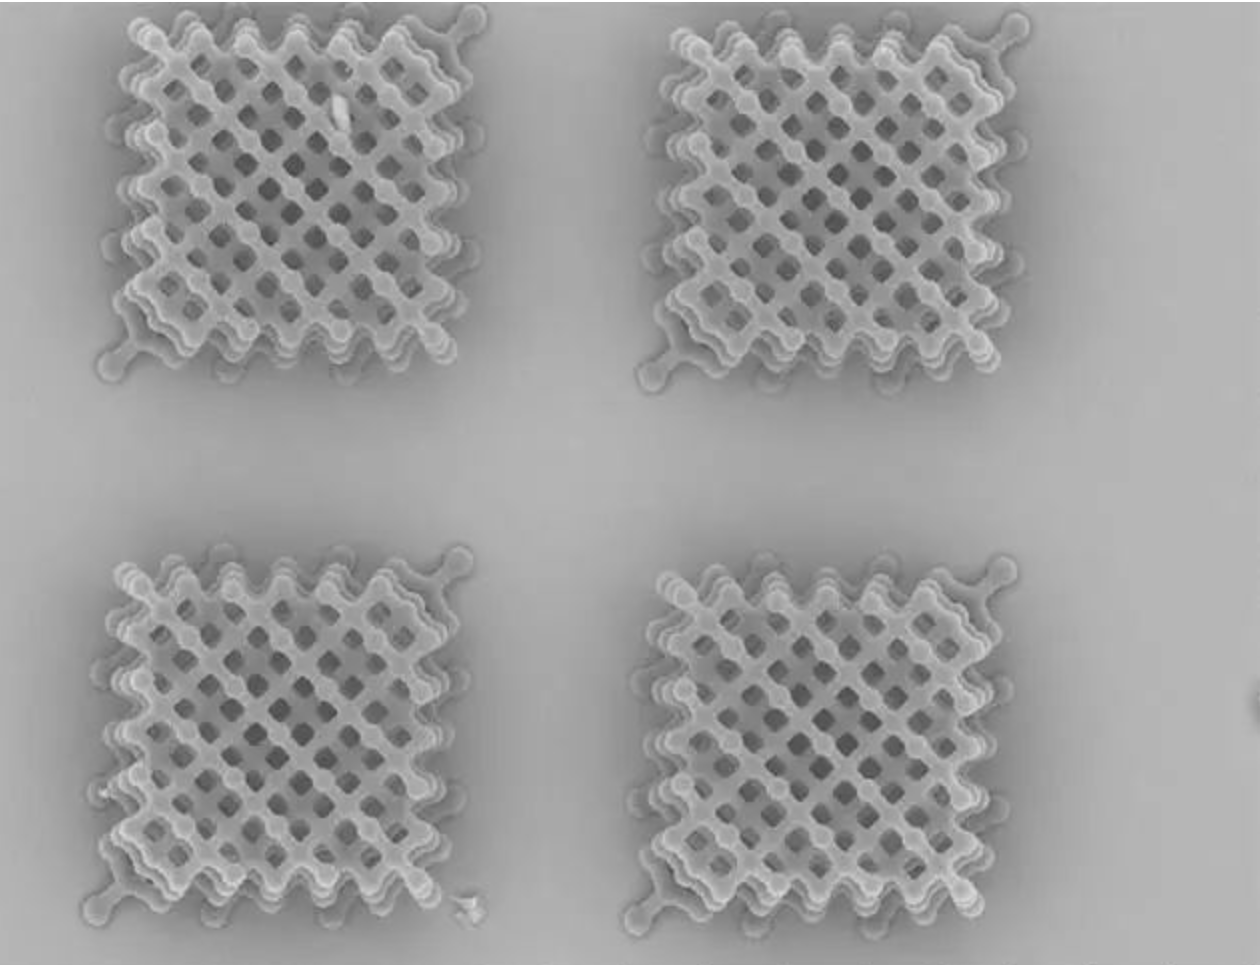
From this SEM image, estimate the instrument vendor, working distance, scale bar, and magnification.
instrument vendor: Hitachi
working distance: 9.5 mm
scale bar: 100000 nm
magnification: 1 K X